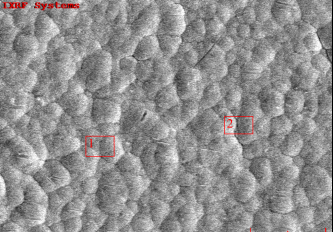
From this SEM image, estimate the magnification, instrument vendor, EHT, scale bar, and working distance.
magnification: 3 K X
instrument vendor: other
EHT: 15 kV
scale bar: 10000 nm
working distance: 10.1 mm